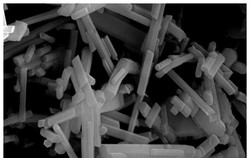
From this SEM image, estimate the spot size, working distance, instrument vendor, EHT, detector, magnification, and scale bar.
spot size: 4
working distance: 5 mm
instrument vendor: FEI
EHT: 20 kV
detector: SE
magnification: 10 K X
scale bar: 5000 nm